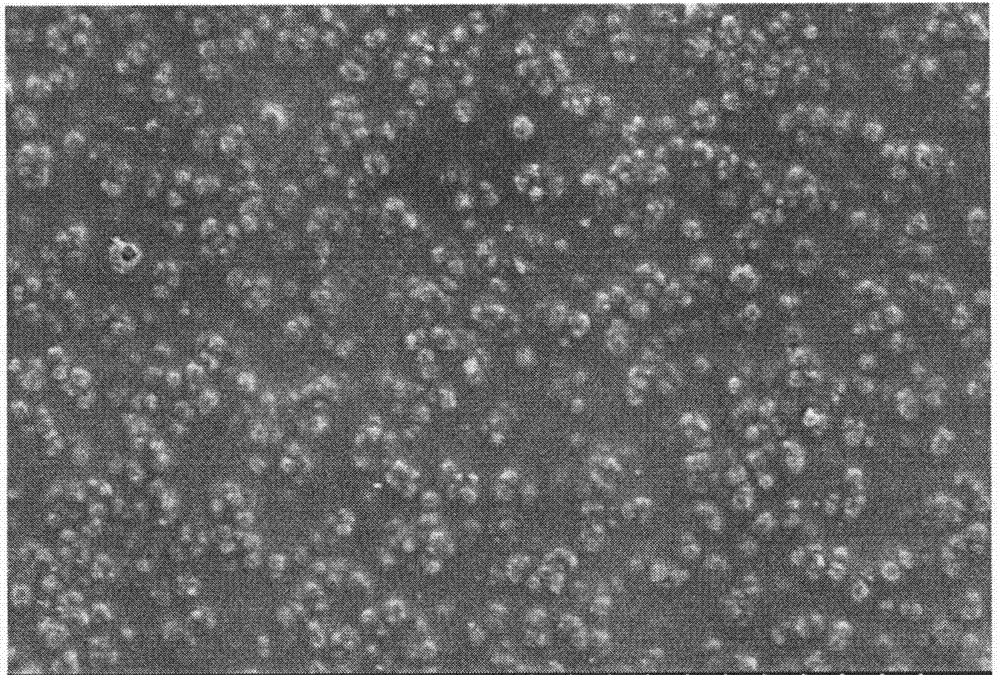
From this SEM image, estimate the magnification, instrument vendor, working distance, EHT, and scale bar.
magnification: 0.5 K X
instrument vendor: Hitachi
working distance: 7 mm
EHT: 10 kV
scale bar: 100000 nm